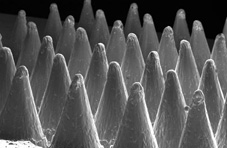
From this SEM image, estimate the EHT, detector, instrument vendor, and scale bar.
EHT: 25 kV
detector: SE1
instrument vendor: Zeiss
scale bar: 1000000 nm